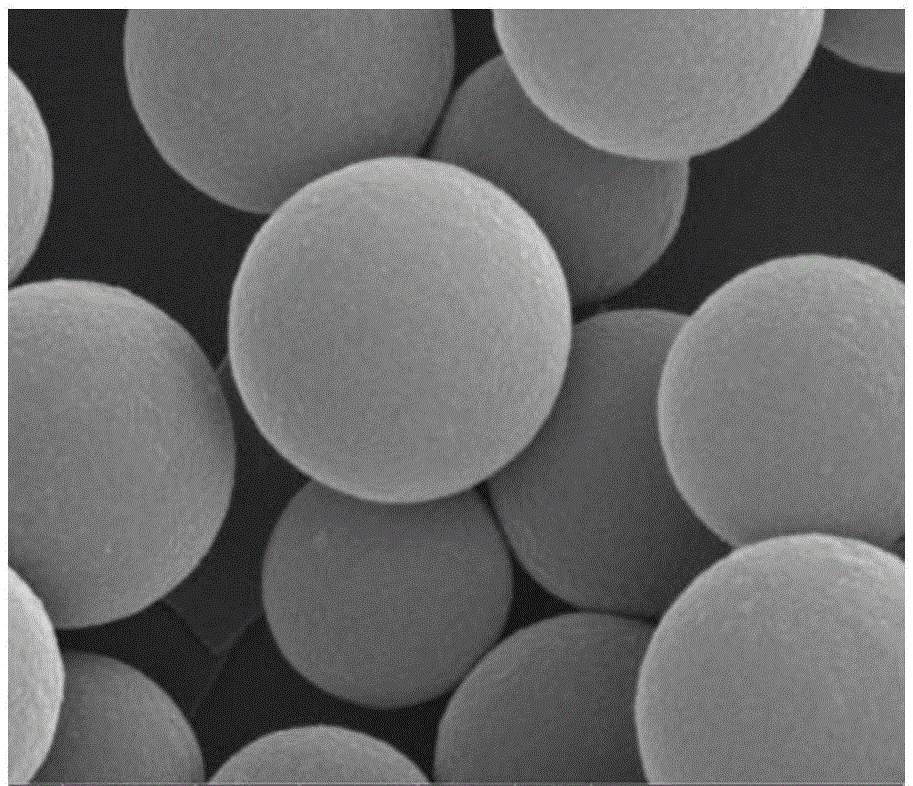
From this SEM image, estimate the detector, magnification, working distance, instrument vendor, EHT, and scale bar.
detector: SE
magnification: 100 K X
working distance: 5.2 mm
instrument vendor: FEI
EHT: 20 kV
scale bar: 500 nm